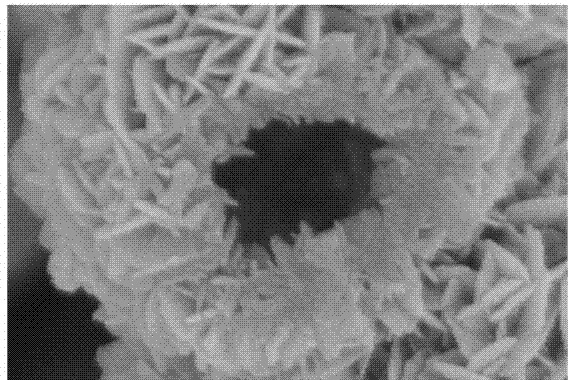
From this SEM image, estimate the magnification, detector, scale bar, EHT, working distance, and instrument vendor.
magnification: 100 K X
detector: SE(M)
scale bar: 500 nm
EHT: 5 kV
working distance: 9.1 mm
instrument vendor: Hitachi